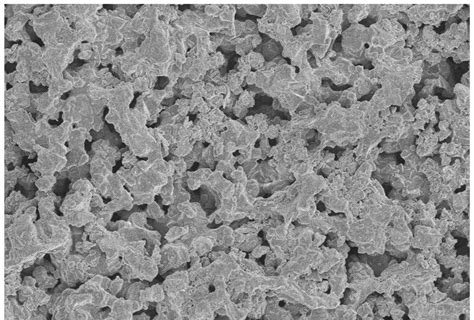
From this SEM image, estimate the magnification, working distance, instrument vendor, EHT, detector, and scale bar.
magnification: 1 K X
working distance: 15.7 mm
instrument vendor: Hitachi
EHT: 5 kV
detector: SE(M)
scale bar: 50000 nm